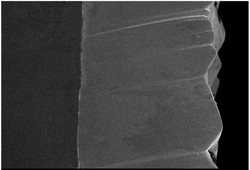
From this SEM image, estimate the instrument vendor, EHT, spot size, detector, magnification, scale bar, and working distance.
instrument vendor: JEOL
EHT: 10 kV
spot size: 40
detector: SEI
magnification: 0.3 K X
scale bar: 50000 nm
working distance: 11 mm